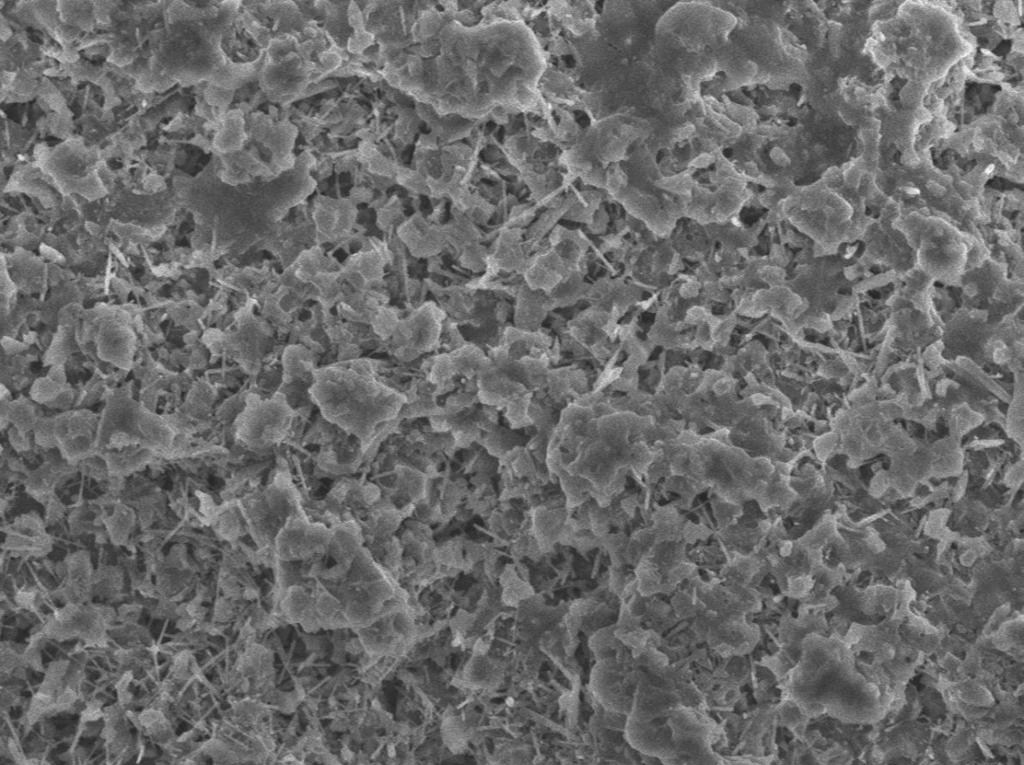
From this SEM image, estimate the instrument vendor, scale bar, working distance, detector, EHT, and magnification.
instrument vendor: JEOL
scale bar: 1000 nm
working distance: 10 mm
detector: SEI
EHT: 5 kV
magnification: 8 K X